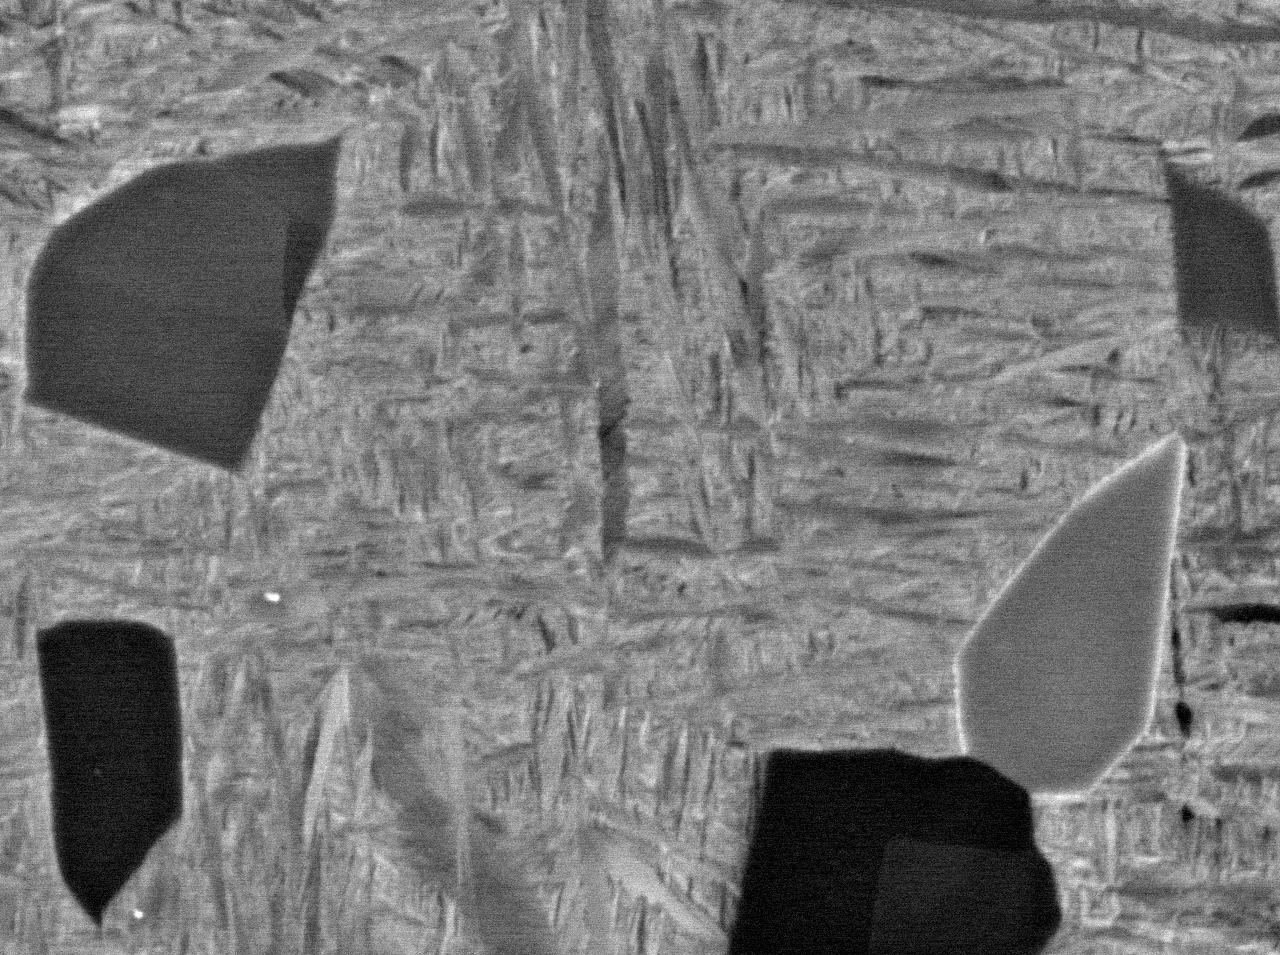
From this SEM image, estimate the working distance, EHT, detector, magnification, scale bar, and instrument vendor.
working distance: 10 mm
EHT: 15 kV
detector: COMPO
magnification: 5 K X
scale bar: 1000 nm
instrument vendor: JEOL